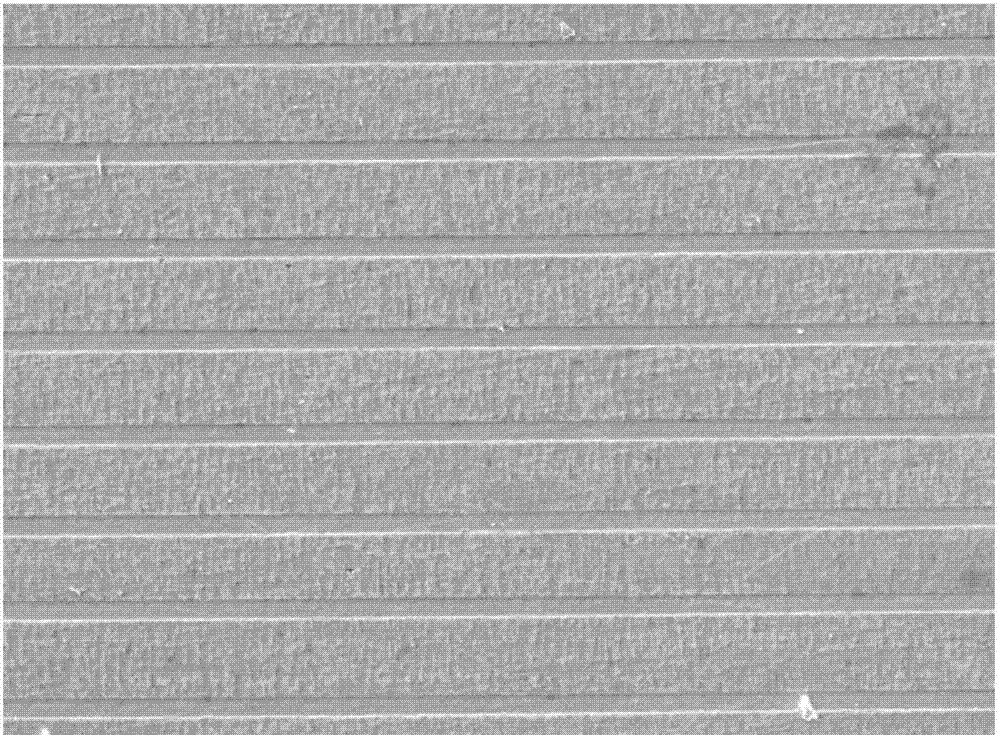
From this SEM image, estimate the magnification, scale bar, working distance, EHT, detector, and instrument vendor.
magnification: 1 K X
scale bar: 10000 nm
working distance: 15.4 mm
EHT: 10 kV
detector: LED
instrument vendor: JEOL